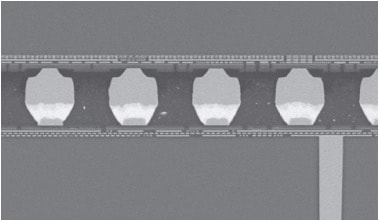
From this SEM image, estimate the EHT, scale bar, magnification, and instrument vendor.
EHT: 25 kV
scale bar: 20000 nm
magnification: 0.6 K X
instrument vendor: JEOL